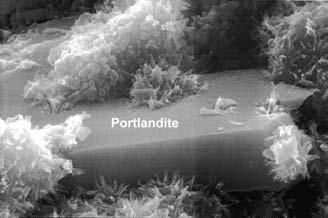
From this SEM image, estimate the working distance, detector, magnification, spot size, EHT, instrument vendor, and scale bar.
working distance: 10.1 mm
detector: GSE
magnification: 16 K X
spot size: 3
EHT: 15 kV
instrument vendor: FEI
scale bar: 2000 nm